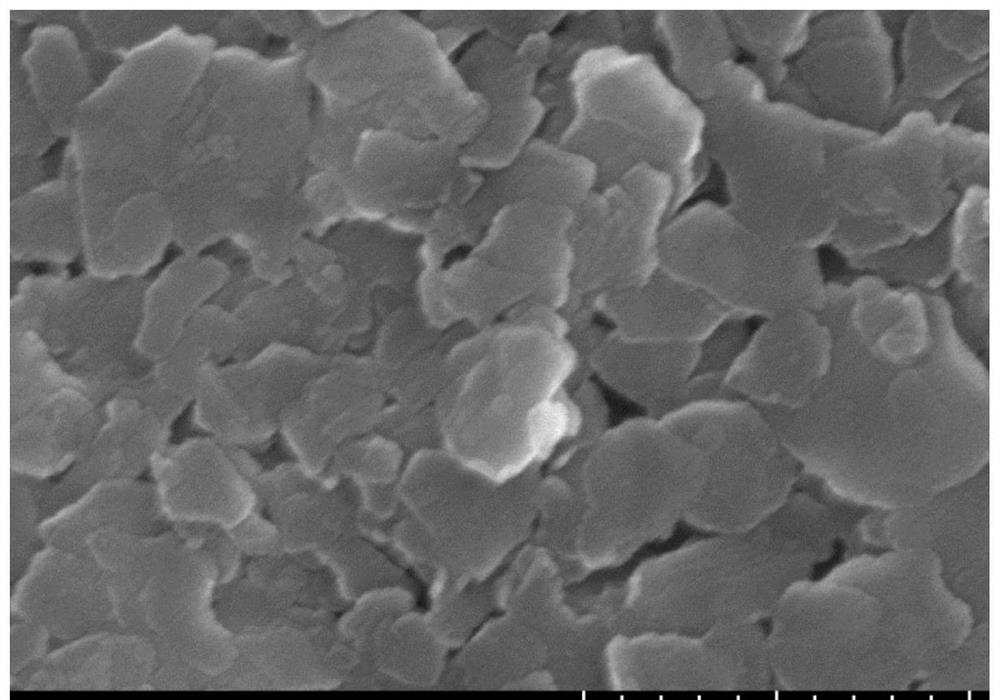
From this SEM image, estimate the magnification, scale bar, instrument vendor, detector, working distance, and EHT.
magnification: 100 K X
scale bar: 500 nm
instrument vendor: Hitachi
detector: SE(UL)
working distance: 9.3 mm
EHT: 10 kV